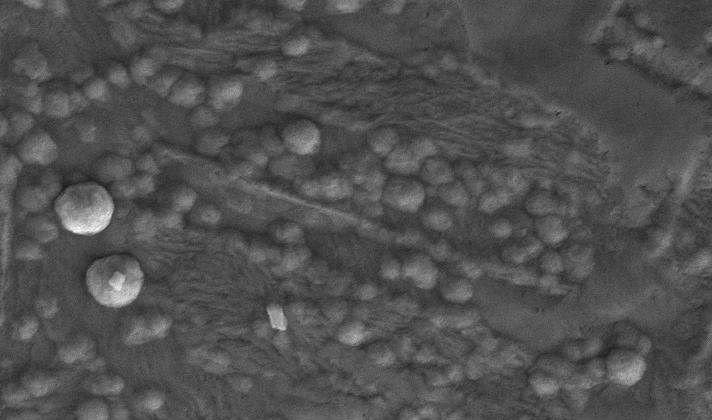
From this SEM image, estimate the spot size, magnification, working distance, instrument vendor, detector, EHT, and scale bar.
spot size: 3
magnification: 8 K X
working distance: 17.6 mm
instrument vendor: FEI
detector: SE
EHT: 20 kV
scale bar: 5000 nm